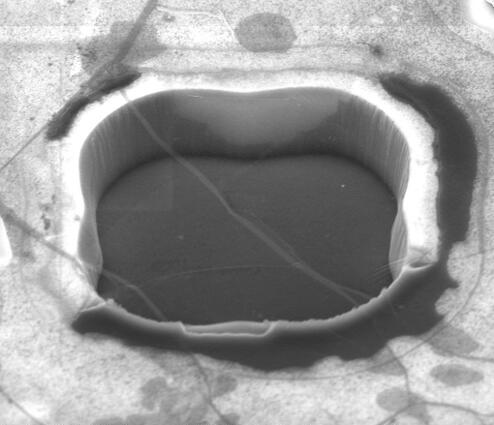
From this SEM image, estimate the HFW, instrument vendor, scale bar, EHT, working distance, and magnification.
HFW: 10.3 µm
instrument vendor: FEI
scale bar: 3000 nm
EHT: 5 kV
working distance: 5.2 mm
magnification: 24.965 K X